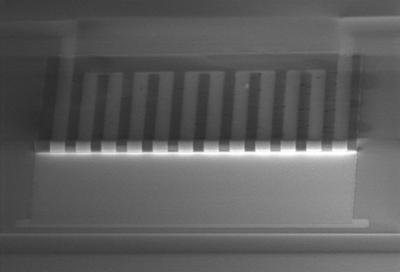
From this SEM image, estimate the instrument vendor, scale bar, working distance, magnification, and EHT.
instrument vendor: Hitachi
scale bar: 100000 nm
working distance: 18.7 mm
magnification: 0.4 K X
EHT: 15 kV